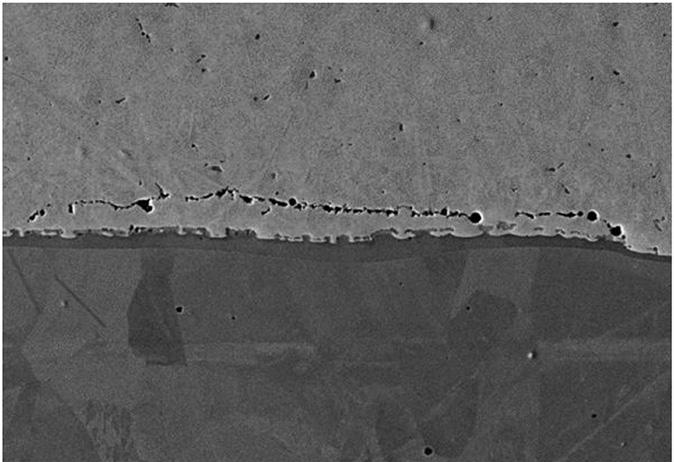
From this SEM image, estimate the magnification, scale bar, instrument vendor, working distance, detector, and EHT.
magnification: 2 K X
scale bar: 10000 nm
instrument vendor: Zeiss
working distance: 13 mm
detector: SE2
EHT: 20 kV